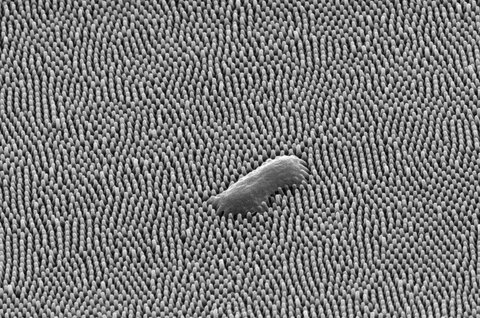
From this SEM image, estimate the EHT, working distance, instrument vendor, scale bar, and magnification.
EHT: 10 kV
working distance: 12 mm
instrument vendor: Thermo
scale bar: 3000 nm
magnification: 20 K X